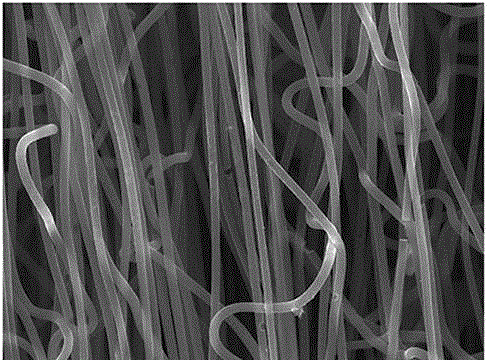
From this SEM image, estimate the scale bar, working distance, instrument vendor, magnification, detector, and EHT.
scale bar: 1000 nm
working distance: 11.1 mm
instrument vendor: JEOL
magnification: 5 K X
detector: SEI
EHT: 15 kV